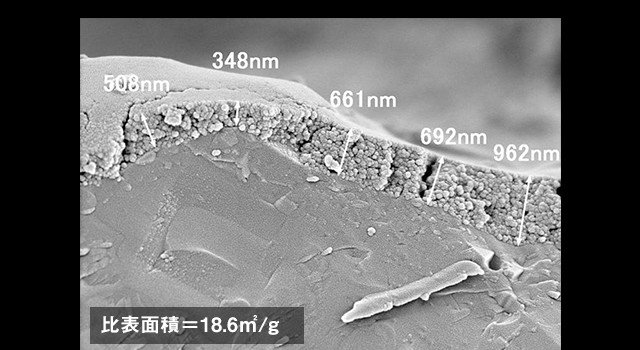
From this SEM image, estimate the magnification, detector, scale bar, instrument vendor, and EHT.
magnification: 20 K X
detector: SE(M)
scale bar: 2000 nm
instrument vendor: Hitachi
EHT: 2 kV